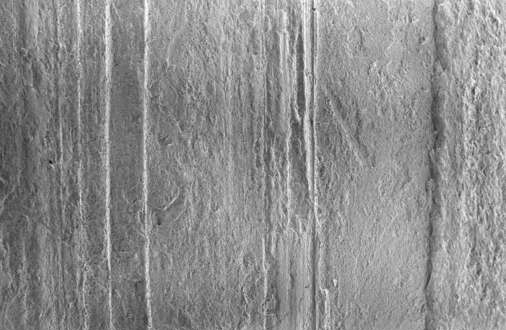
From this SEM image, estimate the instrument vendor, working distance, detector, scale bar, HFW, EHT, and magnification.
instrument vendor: Thermo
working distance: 10.6 mm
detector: ETD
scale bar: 500000 nm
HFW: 1410 µm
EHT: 1 kV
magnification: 0.09 K X